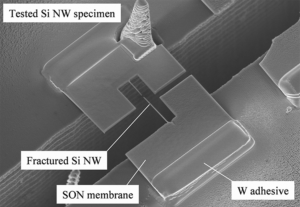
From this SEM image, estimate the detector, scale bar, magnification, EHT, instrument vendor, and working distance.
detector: SE(M,LA0)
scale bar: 10000 nm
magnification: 5 K X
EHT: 5 kV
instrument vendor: Hitachi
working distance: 7.9 mm